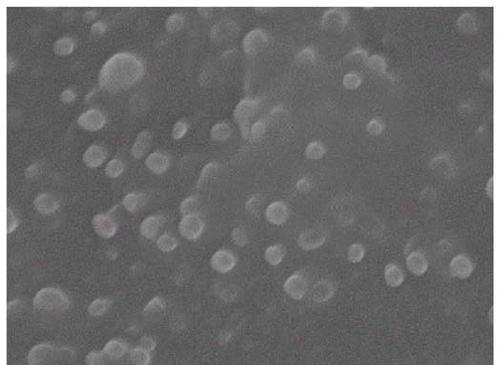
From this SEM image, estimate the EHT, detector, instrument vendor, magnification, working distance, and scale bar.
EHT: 10 kV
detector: SEI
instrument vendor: JEOL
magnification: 50 K X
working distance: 6.5 mm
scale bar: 100 nm